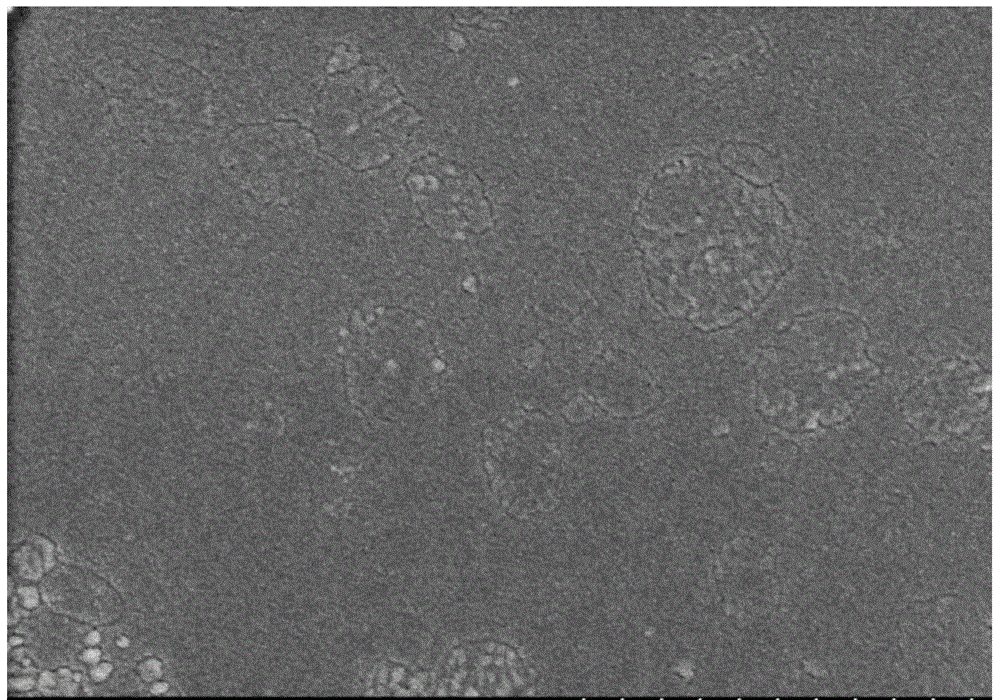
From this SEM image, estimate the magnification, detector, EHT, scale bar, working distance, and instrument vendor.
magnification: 50 K X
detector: SE(M)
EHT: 3 kV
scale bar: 1000 nm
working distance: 6.2 mm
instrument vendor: Hitachi